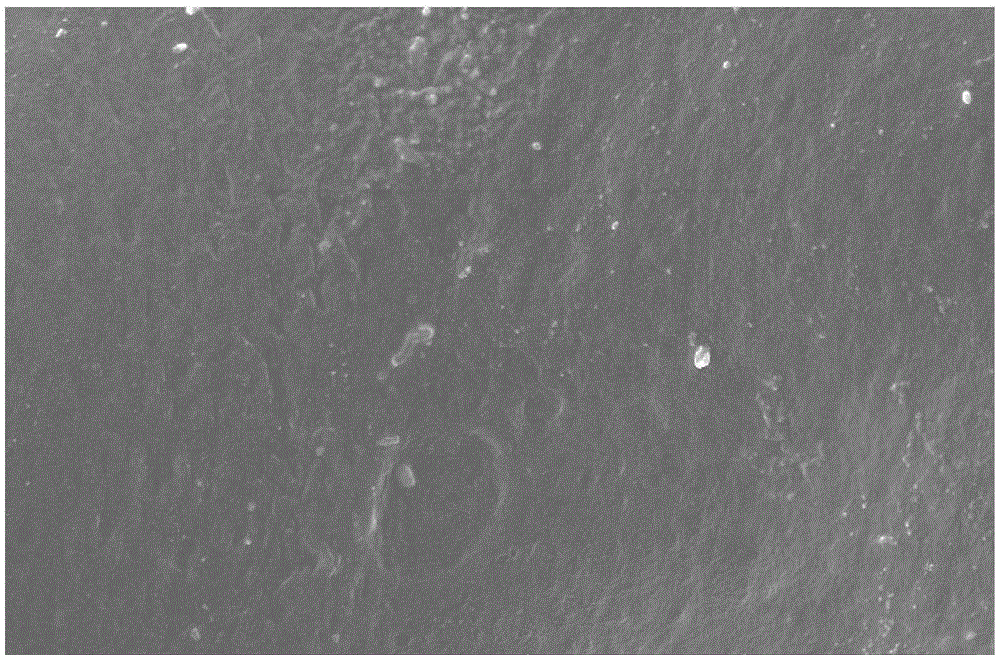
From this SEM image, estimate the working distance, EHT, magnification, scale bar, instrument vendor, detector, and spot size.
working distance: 23 mm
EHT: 10 kV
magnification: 1 K X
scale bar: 20000 nm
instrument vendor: FEI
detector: SE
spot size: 3